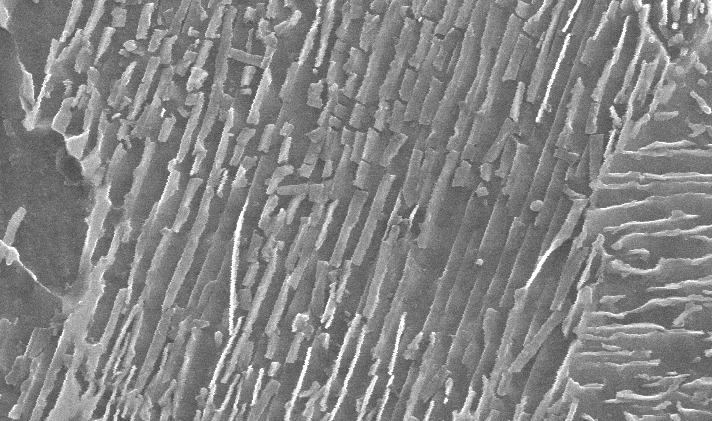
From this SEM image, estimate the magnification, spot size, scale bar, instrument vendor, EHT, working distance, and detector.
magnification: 20 K X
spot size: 3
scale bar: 1000 nm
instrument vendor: FEI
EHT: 25 kV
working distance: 9.7 mm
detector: SE